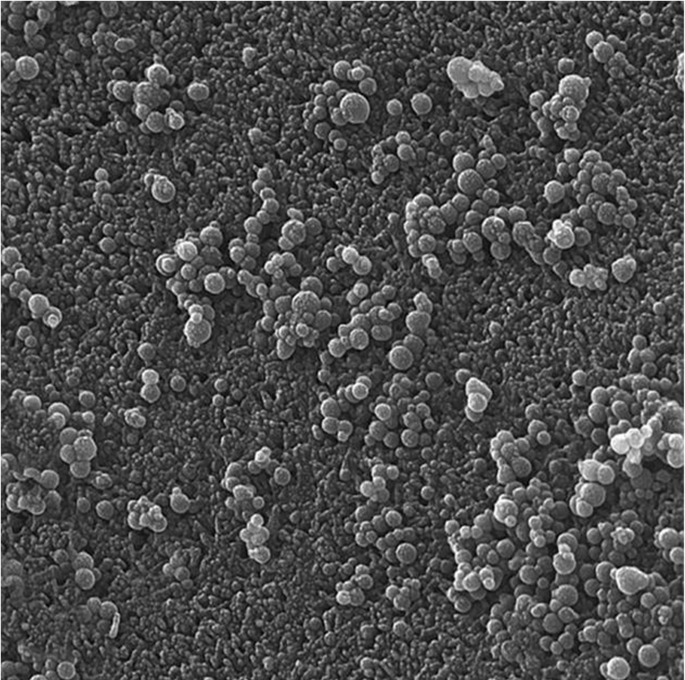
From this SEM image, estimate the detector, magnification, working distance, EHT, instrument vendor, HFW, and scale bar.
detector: In-Beam SE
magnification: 75 K X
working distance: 5.62 mm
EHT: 15 kV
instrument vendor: Tescan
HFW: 2.77 µm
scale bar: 500 nm